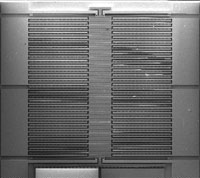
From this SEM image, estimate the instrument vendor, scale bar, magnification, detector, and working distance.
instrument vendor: FEI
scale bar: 500000 nm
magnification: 0.045 K X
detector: SE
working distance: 18.3 mm